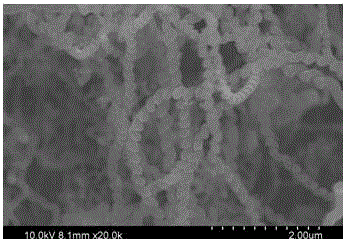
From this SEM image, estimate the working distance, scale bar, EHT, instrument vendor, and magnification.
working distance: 8.1 mm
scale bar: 2000 nm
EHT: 10 kV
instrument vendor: Hitachi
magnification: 20 K X